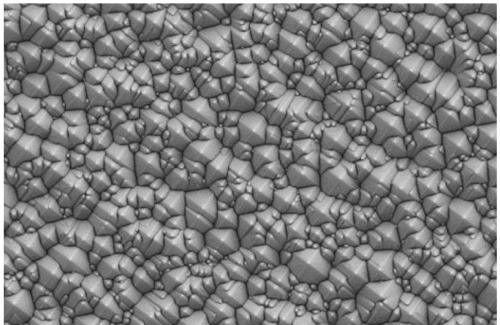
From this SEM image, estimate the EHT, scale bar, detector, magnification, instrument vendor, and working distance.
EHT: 5 kV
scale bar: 2000 nm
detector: SE2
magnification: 2 K X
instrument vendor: Zeiss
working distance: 8.5 mm